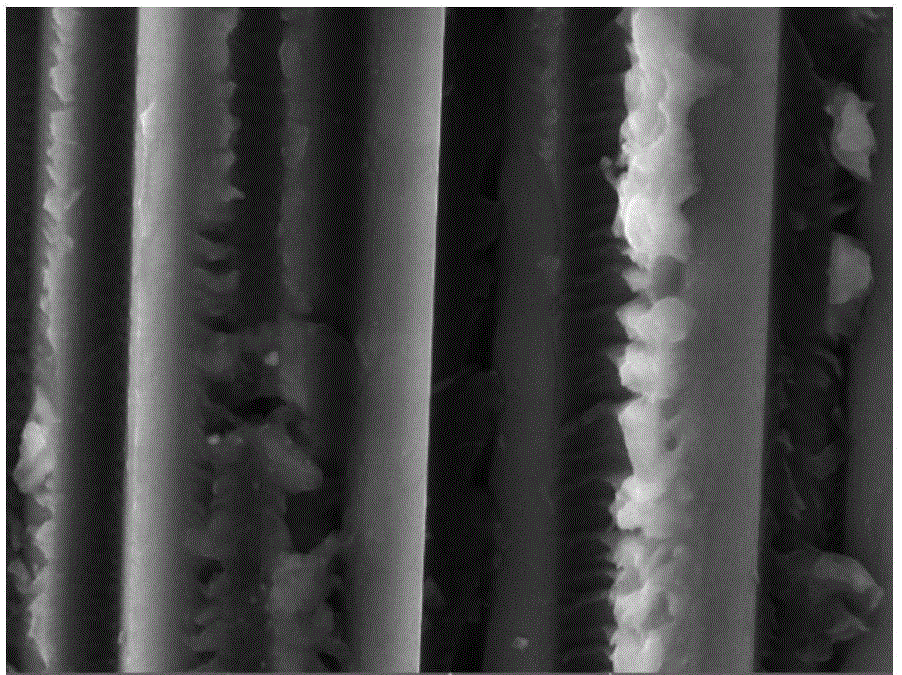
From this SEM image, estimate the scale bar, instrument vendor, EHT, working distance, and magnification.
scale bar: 10000 nm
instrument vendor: Tescan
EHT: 20 kV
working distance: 16.56 mm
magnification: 5 K X